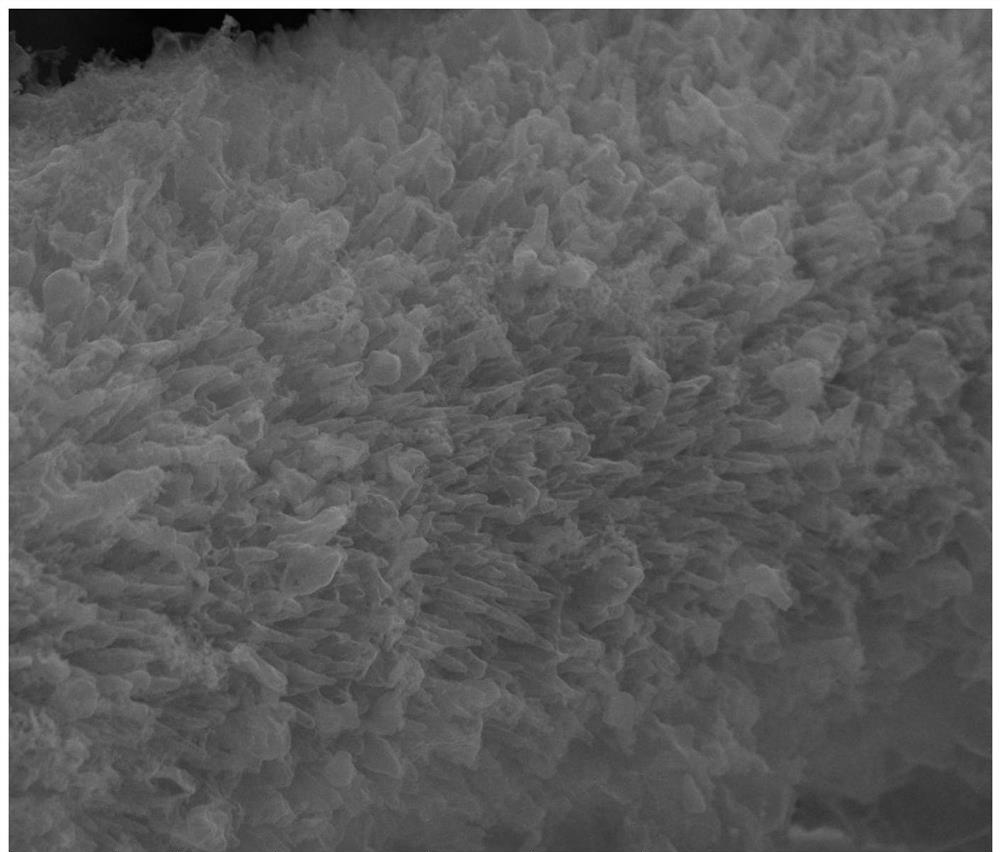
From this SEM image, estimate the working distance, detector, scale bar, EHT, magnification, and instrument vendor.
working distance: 11.2 mm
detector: ETD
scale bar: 5000 nm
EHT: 20 kV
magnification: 20 K X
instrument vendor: FEI